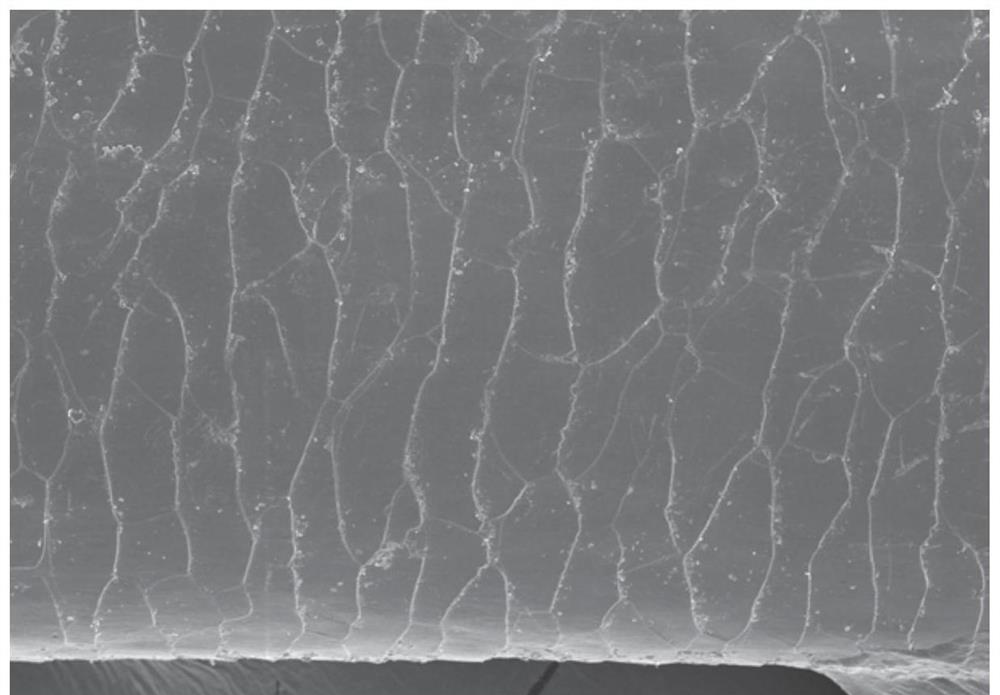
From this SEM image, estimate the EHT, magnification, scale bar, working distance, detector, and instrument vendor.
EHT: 5 kV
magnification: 0.5 K X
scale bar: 100000 nm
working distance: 14.9 mm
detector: SE(M)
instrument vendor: Hitachi